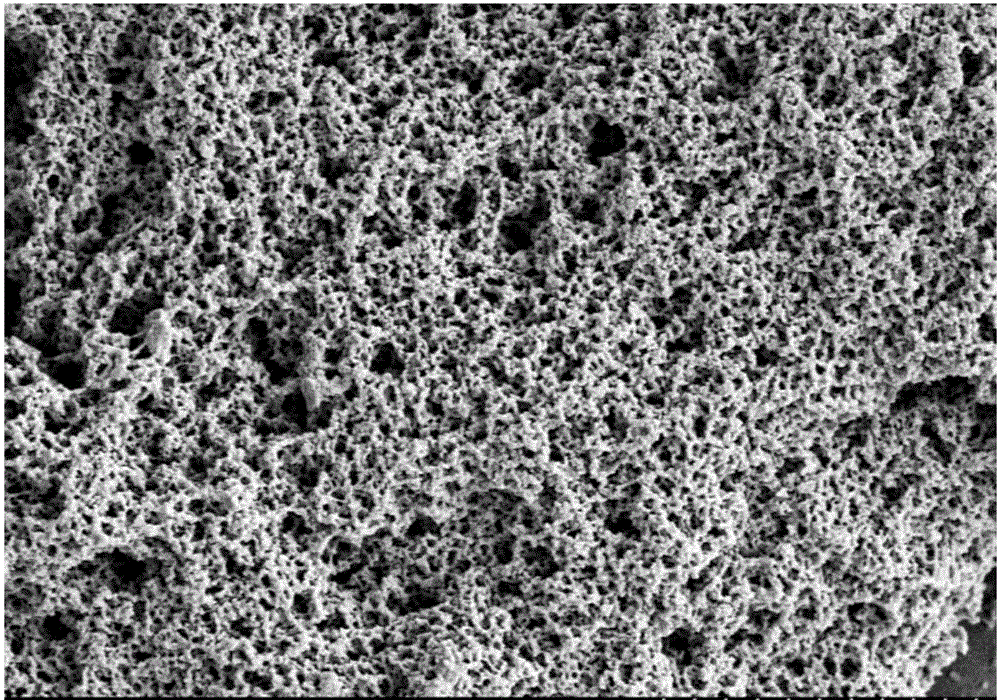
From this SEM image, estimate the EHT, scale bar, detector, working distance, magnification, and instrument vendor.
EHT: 5 kV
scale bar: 20000 nm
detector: SE(M,LA0)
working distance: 12.1 mm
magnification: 2 K X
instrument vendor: Hitachi